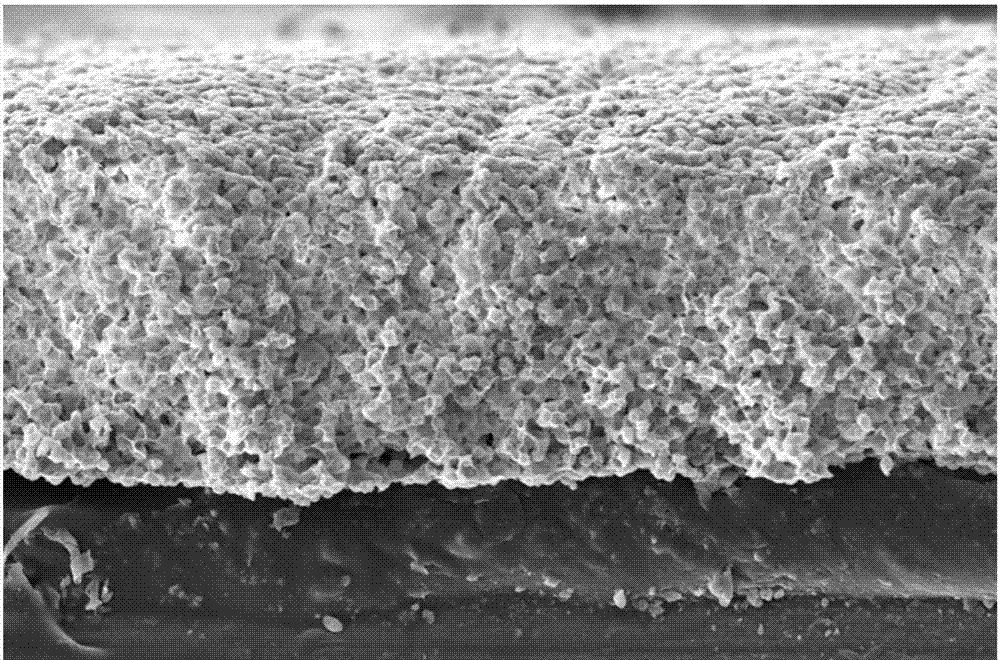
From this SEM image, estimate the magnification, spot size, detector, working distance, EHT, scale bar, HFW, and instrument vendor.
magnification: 1 K X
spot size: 3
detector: ETD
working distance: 8.5 mm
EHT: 20 kV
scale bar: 100000 nm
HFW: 414 µm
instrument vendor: FEI